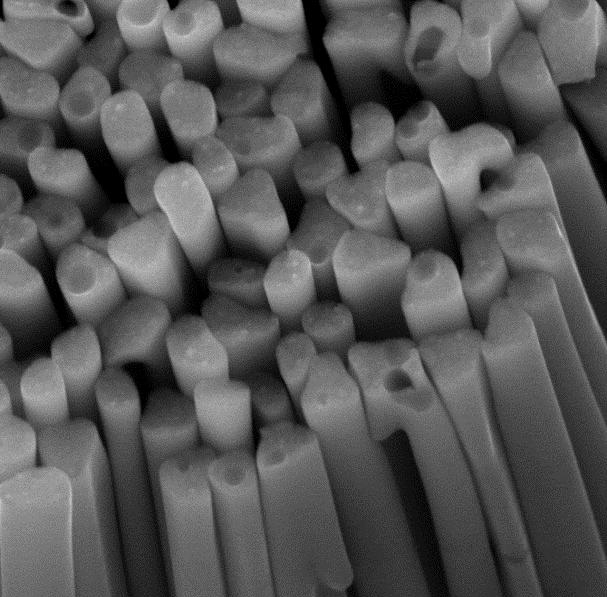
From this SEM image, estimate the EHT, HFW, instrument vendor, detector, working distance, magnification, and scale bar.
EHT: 15 kV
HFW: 2.167 µm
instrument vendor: Tescan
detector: InBeam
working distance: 2.477 mm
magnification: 100 K X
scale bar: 500 nm